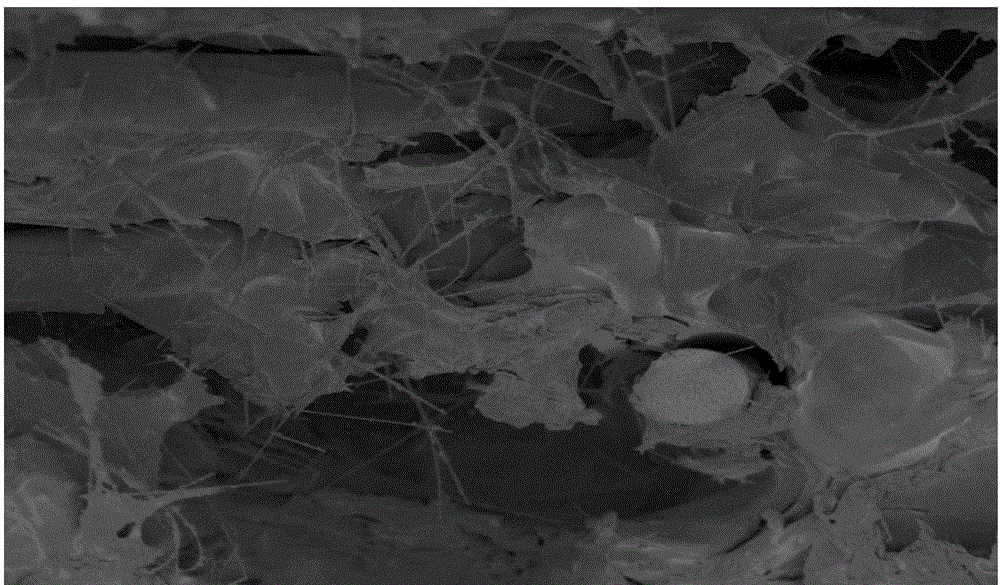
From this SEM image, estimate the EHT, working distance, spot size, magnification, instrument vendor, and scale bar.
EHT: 3 kV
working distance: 6.3 mm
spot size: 3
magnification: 5 K X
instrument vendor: FEI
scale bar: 20000 nm